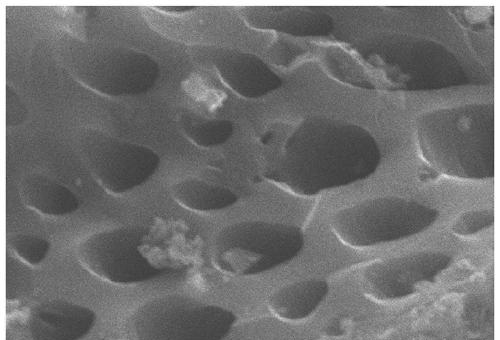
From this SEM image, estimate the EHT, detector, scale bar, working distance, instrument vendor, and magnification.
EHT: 20 kV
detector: SE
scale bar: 10000 nm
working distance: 10 mm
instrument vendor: Hitachi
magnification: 5 K X